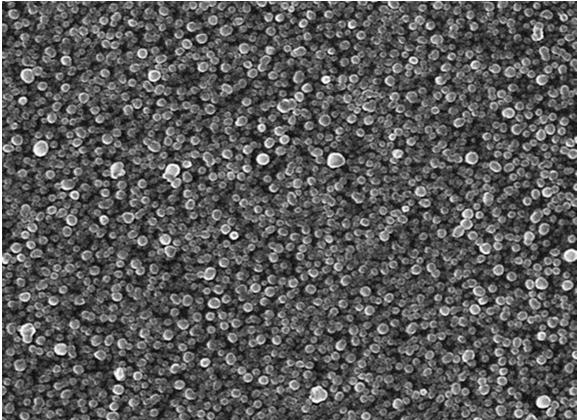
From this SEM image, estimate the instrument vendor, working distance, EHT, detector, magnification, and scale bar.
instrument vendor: JEOL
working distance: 6.5 mm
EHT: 15 kV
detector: SEI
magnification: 50 K X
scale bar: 100 nm